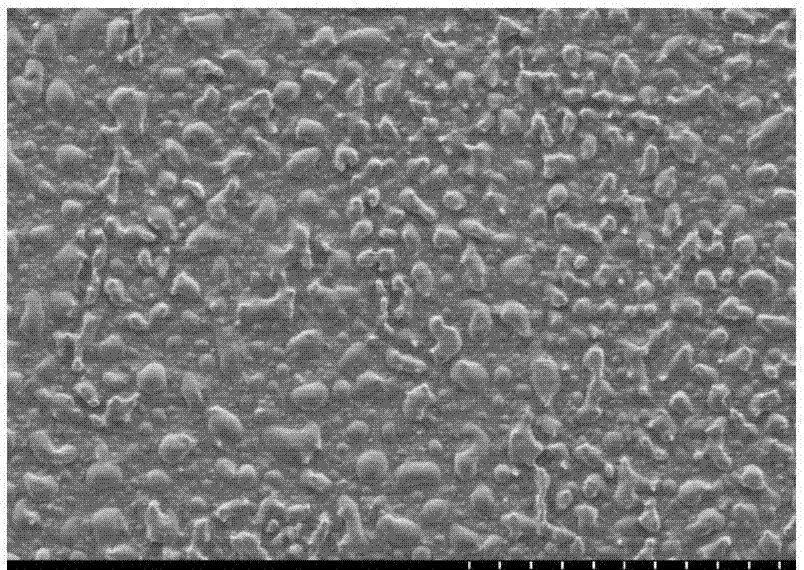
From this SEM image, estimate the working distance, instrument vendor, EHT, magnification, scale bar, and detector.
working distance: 7.7 mm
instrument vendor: Hitachi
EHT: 5 kV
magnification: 1 K X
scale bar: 50000 nm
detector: SE(M)